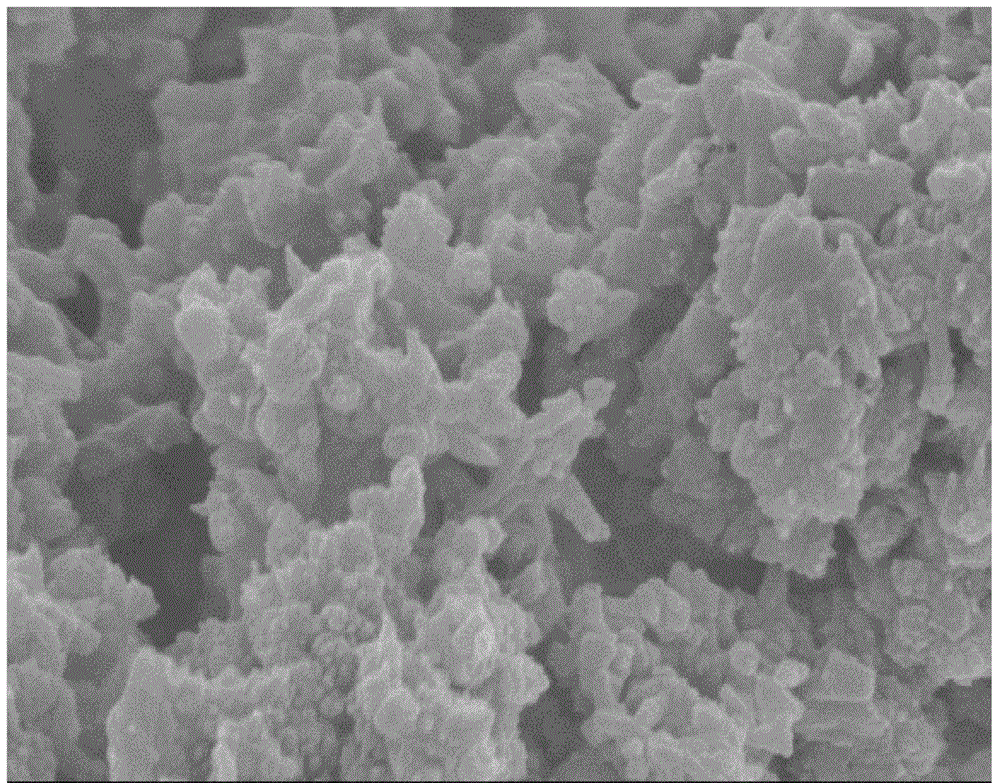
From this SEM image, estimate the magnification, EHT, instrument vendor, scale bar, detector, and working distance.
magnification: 50 K X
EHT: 3 kV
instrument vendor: Hitachi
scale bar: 1000 nm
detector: SE(M)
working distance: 8.5 mm